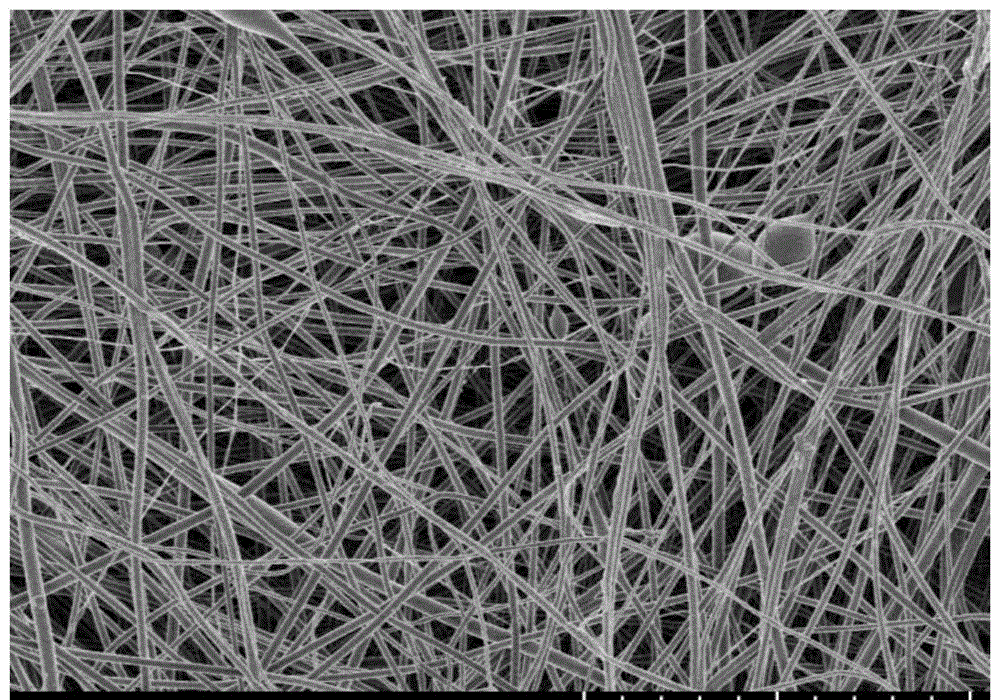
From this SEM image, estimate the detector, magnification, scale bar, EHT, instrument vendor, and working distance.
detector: SE(U)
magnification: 5 K X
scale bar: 10000 nm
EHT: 1.5 kV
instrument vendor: Hitachi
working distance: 3.3 mm